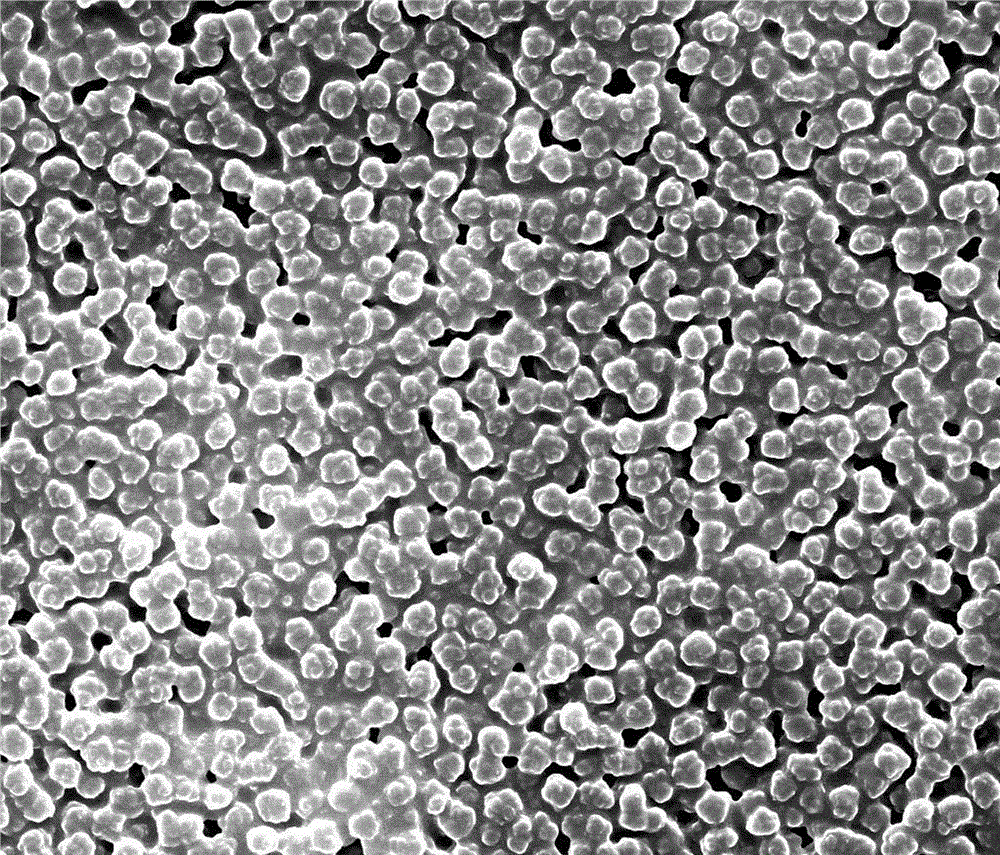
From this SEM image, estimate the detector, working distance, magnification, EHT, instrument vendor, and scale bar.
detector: SE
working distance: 11.6 mm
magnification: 20 K X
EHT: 20 kV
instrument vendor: FEI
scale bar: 5000 nm